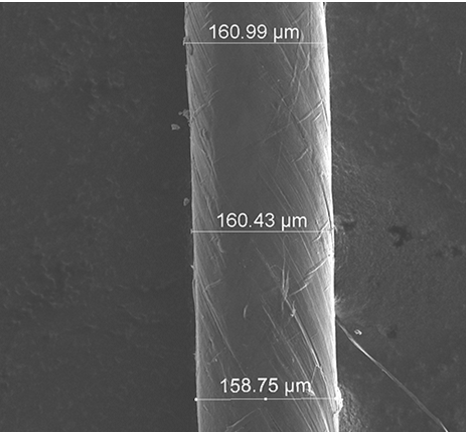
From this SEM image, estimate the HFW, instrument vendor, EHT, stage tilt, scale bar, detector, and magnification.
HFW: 574 µm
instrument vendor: FEI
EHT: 10 kV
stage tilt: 0°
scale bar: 200000 nm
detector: ETD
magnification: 0.25 K X